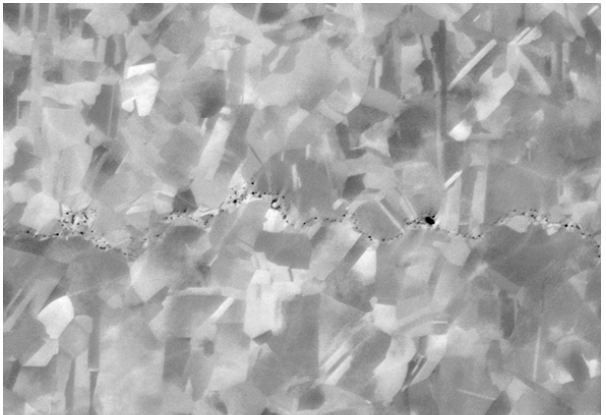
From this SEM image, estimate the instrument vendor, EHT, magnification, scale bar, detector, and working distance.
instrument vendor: Zeiss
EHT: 8 kV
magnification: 8 K X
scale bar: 1000 nm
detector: NTS BSD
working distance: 6.5 mm